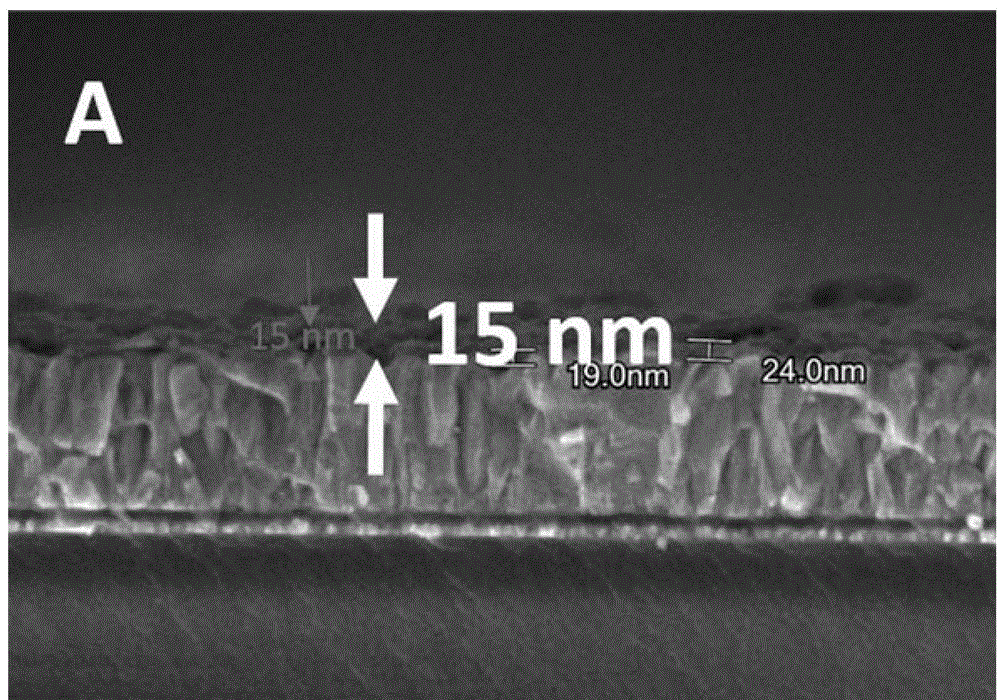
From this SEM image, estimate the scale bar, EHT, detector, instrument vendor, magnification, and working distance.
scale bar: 500 nm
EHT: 3 kV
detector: SE(U)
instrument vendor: Hitachi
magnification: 100 K X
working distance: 1.9 mm